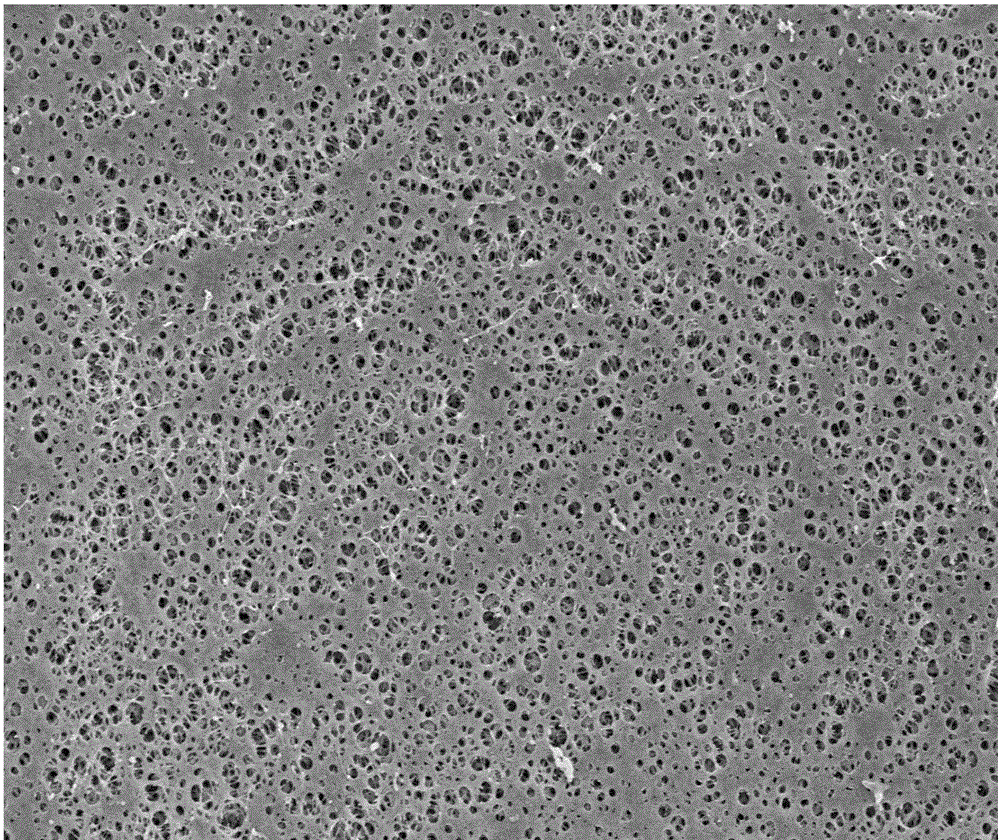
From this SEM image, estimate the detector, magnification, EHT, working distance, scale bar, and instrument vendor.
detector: SE(M)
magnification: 3 K X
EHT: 5 kV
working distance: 10.9 mm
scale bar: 10000 nm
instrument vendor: Hitachi